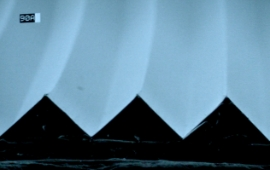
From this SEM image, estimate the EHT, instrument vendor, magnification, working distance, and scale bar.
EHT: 20 kV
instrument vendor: other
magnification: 0.15 K X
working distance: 12 mm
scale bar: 100000 nm